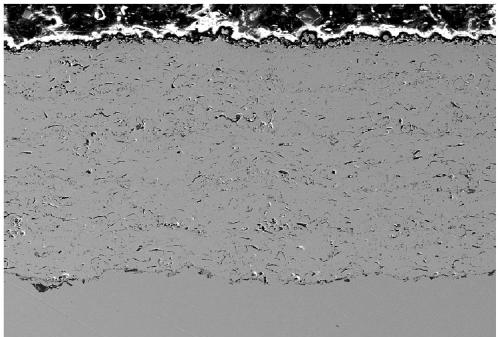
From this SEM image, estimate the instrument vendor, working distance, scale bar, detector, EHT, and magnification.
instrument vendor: Zeiss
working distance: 8.8 mm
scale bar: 100000 nm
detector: SE2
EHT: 15 kV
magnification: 0.15 K X